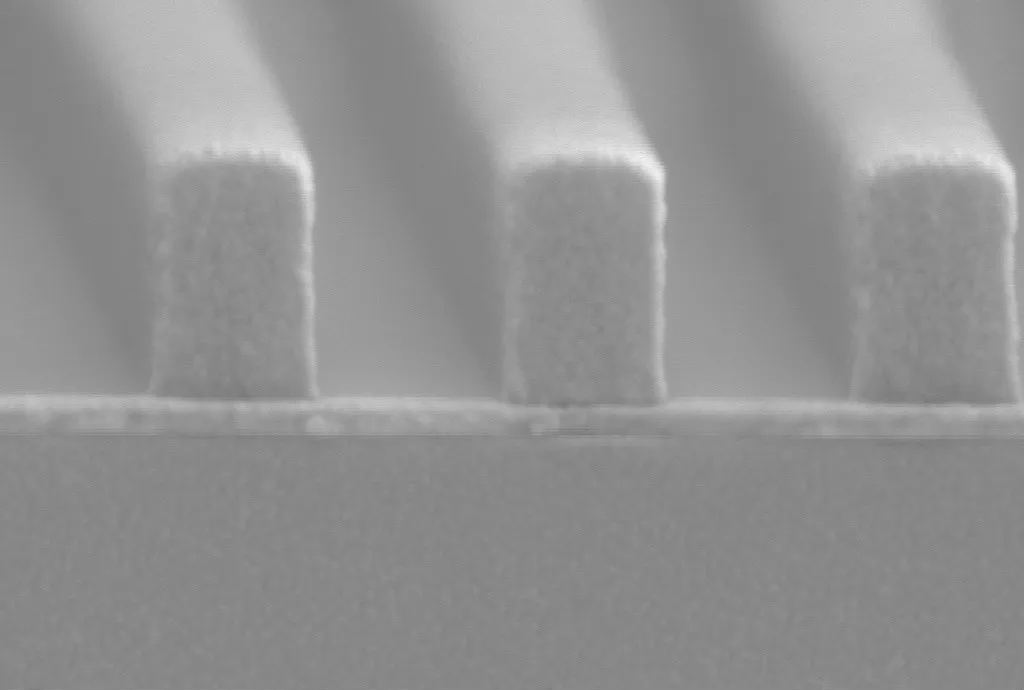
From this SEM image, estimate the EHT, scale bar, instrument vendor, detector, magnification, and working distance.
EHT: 3 kV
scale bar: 100 nm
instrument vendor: Zeiss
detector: SE2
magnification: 160 K X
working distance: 7.7 mm